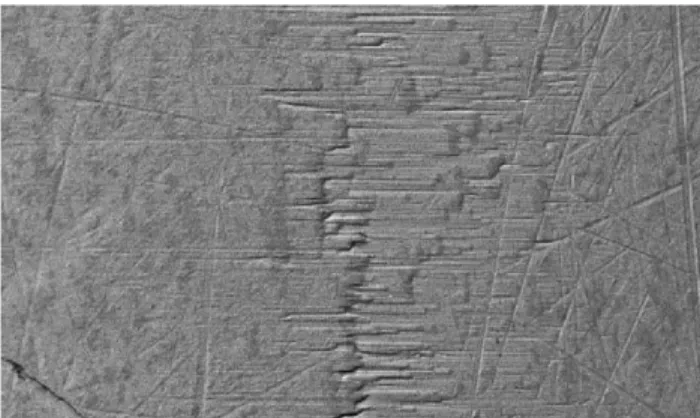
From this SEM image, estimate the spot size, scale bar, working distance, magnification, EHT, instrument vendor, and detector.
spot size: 4.6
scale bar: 100000 nm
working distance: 10 mm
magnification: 0.25 K X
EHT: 20 kV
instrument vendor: FEI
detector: BSE